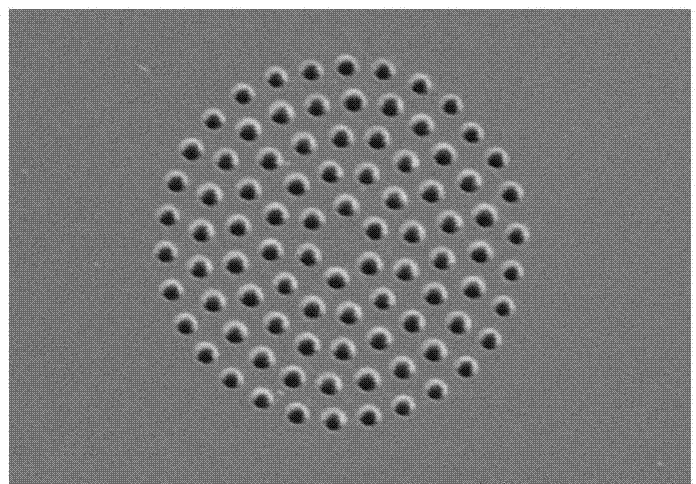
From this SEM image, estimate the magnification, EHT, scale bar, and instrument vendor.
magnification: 2 K X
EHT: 5 kV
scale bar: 20000 nm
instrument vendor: Hitachi